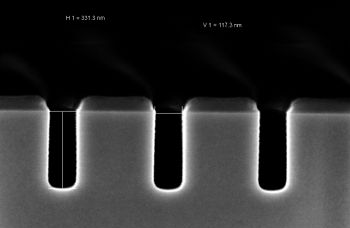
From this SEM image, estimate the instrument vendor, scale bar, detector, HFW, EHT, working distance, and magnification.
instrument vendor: Zeiss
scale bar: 100 nm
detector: InLens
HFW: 1.501 µm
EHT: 2 kV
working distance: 3.3 mm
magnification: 200 K X